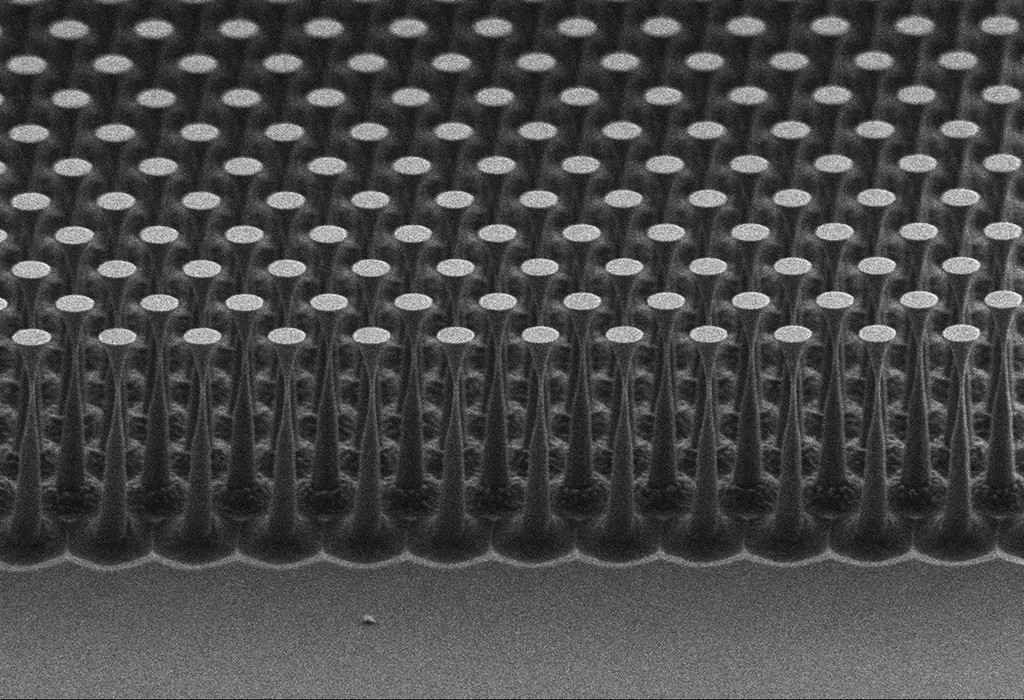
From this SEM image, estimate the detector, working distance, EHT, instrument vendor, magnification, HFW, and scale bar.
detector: SE2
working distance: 6.6 mm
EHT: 2 kV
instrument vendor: Zeiss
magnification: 21.92 K X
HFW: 13.68 µm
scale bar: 1000 nm